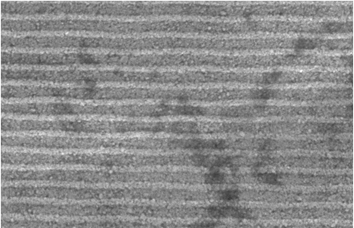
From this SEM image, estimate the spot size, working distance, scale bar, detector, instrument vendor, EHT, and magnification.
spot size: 4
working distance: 6.7 mm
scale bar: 2000 nm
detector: GSE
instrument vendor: FEI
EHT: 20 kV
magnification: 8.41 K X